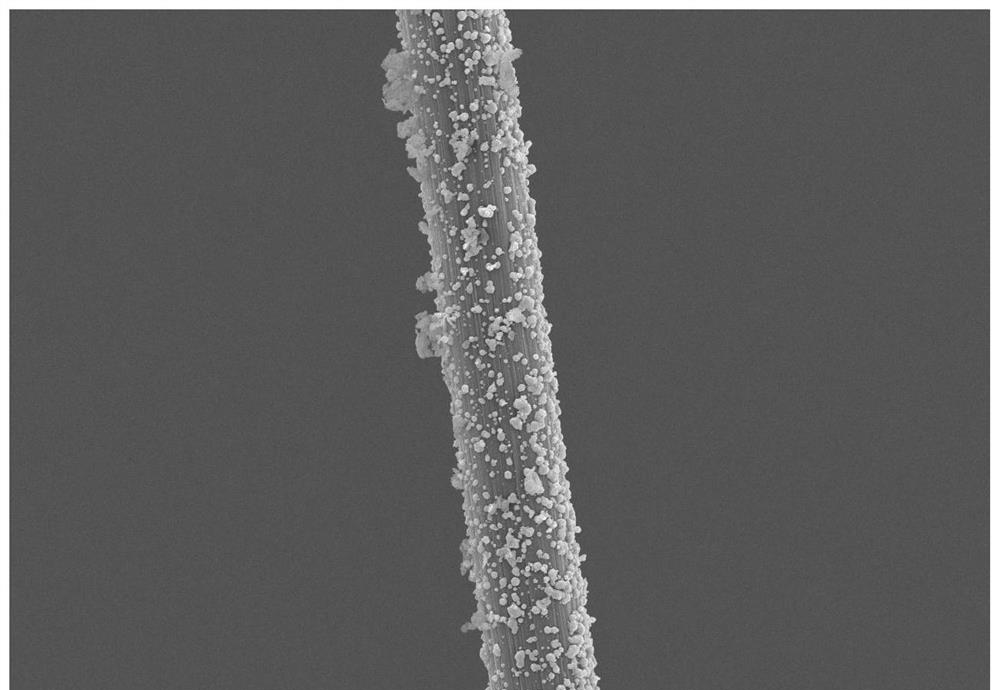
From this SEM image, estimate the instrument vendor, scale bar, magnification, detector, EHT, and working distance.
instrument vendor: Hitachi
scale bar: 20000 nm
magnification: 2 K X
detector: SE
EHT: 15 kV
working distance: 9.4 mm